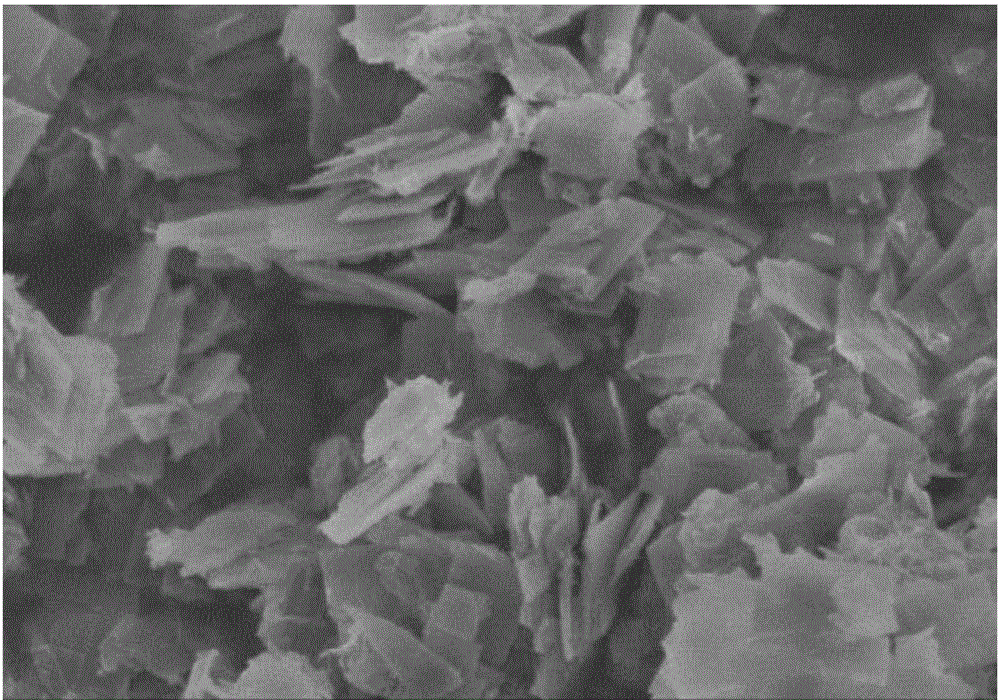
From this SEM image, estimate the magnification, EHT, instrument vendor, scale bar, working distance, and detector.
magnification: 25 K X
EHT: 3 kV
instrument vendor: Hitachi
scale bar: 2000 nm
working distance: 10.7 mm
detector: SE(M)